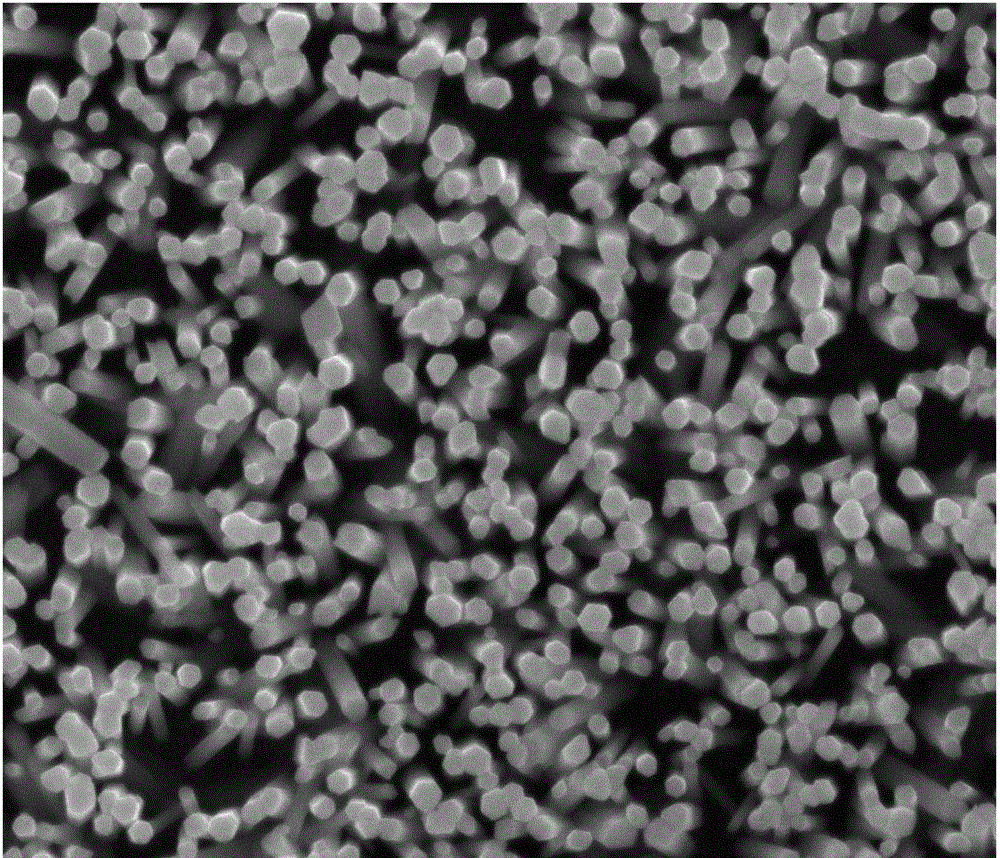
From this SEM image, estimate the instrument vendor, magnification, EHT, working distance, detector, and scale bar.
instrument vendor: FEI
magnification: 50 K X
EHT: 20 kV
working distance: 10.6 mm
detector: SE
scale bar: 2000 nm